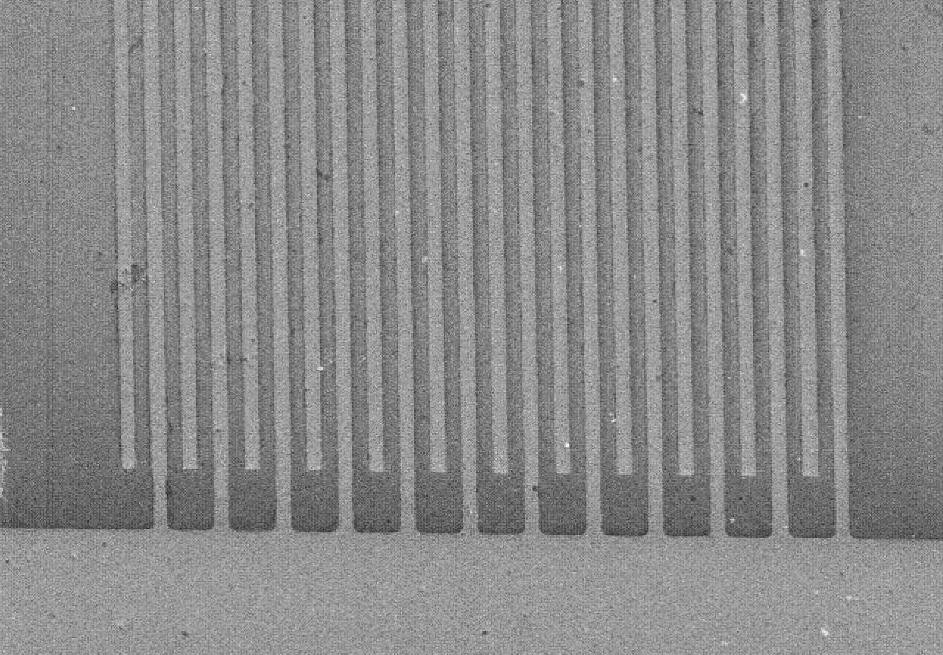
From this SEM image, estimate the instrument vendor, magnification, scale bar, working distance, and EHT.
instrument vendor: Hitachi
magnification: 0.35 K X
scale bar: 100000 nm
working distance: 6 mm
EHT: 5 kV